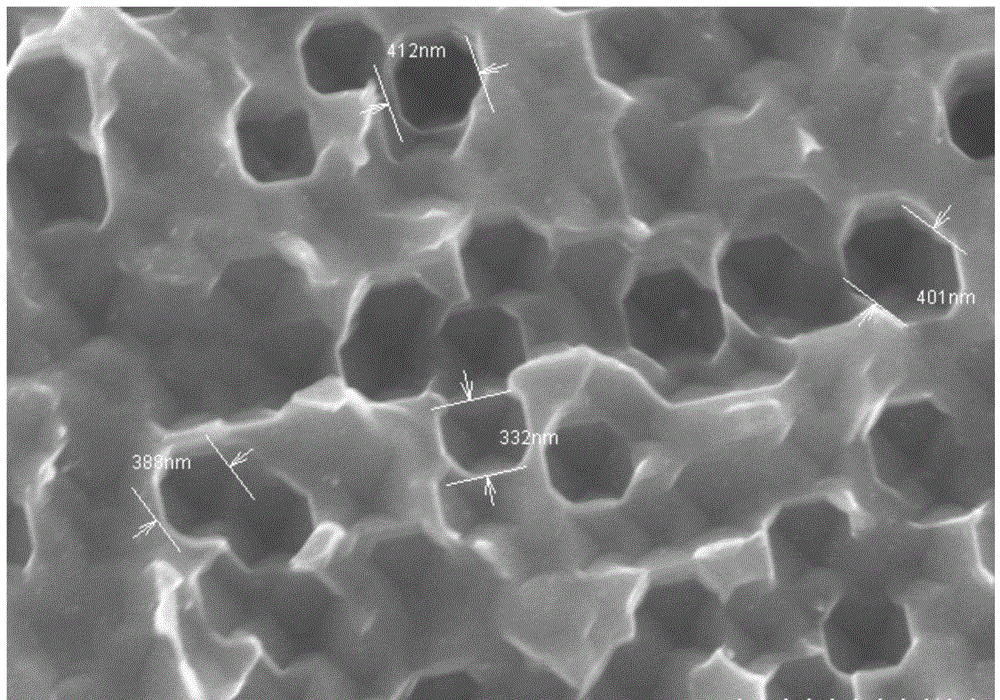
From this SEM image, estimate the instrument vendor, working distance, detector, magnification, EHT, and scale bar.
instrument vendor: Hitachi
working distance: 3.5 mm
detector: SE(M)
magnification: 30 K X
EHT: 10 kV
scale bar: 1000 nm